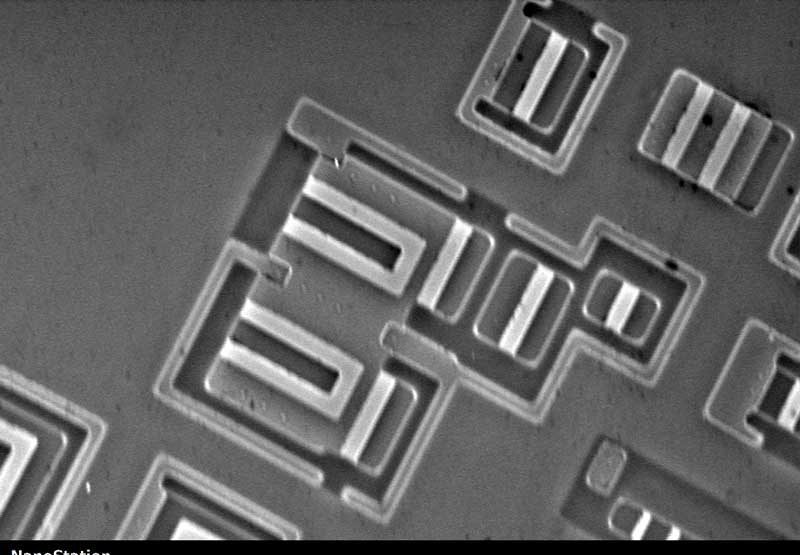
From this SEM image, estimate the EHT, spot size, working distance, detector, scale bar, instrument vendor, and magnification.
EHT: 20 kV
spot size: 14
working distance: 7.7 mm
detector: SE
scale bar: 10000 nm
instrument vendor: other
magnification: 2 K X